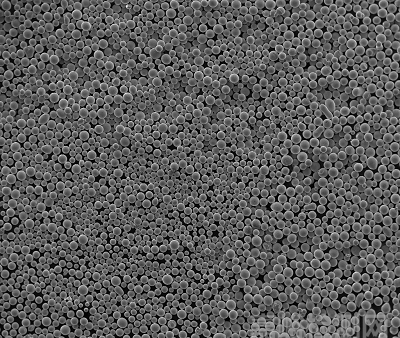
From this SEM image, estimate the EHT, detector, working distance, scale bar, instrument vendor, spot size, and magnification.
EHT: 30 kV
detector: ETD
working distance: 11.3 mm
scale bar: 1000000 nm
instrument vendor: FEI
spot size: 6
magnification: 0.1 K X